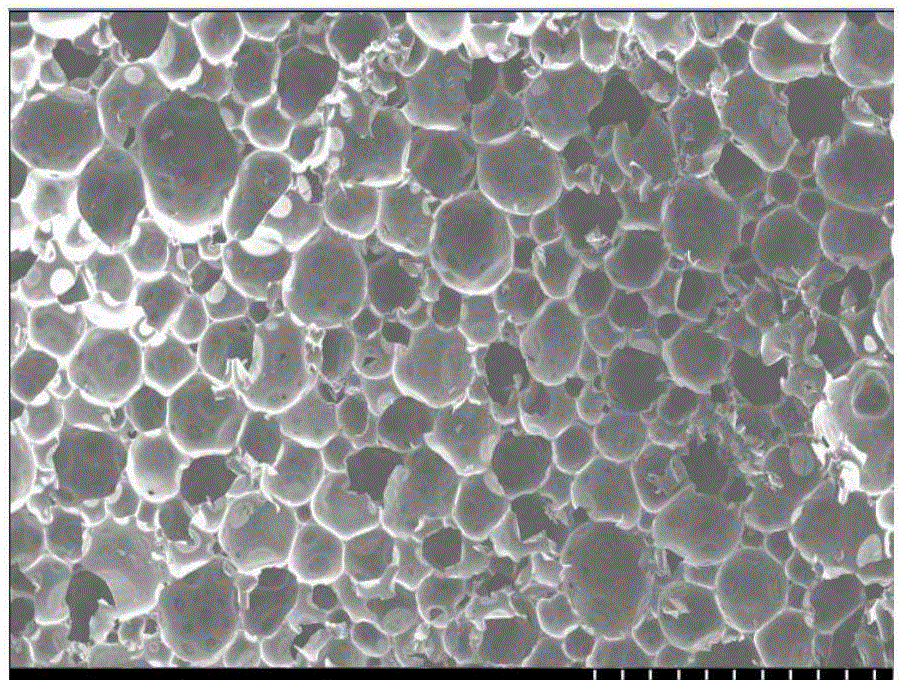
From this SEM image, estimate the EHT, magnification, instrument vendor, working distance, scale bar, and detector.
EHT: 15 kV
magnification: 0.02 K X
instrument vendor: Hitachi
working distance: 23.4 mm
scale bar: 2000000 nm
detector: SE